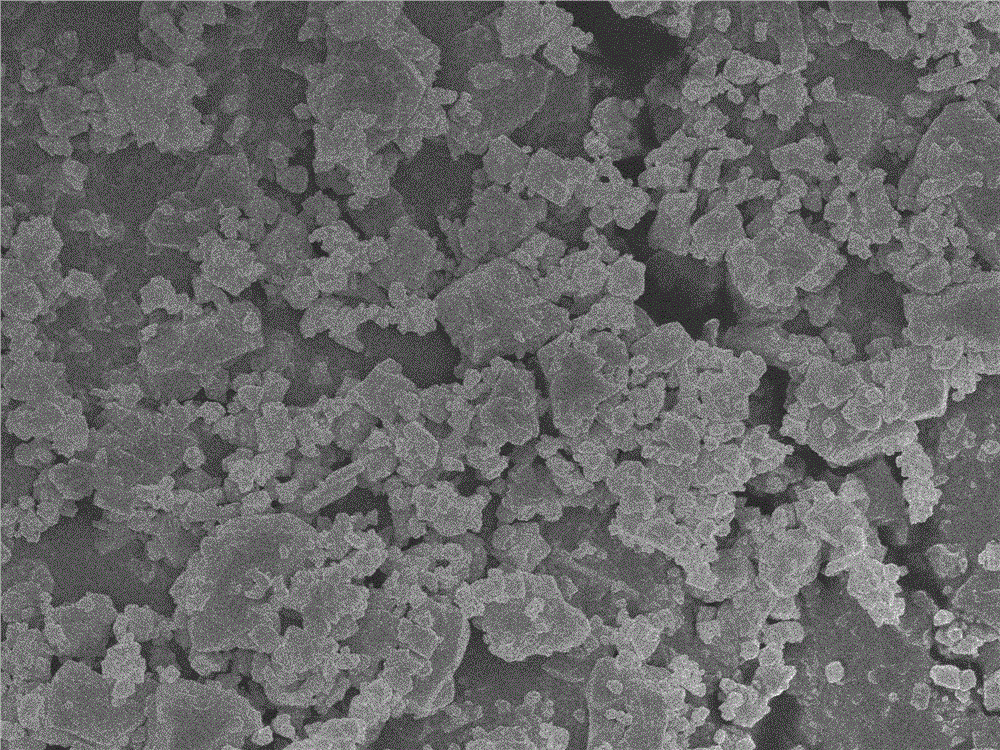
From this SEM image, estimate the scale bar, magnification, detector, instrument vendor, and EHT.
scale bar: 1000 nm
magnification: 10 K X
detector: SEI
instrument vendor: JEOL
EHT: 5 kV